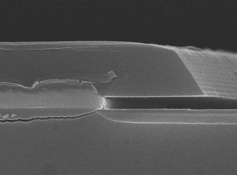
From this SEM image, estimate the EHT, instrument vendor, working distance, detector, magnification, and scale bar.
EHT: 15 kV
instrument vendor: JEOL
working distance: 7.7 mm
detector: SEI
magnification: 20 K X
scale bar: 1000 nm